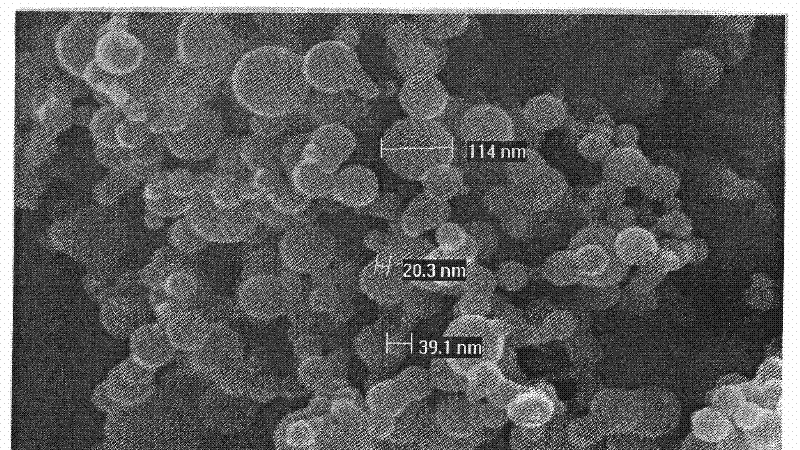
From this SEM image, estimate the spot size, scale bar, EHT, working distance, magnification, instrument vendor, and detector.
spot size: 3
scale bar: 200 nm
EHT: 10 kV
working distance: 4.7 mm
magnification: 250 K X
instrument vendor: FEI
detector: TLD